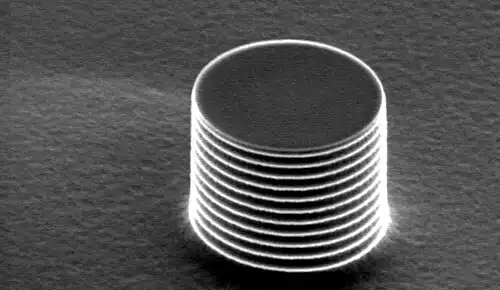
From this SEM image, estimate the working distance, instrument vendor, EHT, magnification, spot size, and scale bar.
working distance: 21.8 mm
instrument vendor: FEI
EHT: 5 kV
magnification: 5 K X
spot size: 3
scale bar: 5000 nm